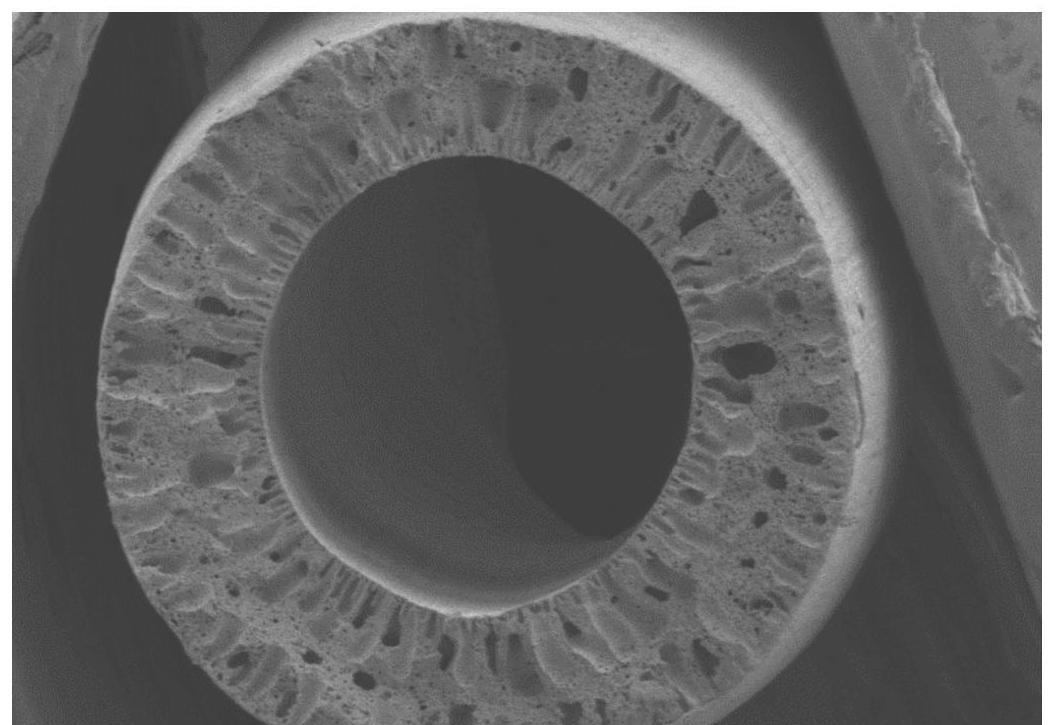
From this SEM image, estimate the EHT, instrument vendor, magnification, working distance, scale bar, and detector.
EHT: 5 kV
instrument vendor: Hitachi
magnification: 0.08 K X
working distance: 6.8 mm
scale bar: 500000 nm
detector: SE(M)